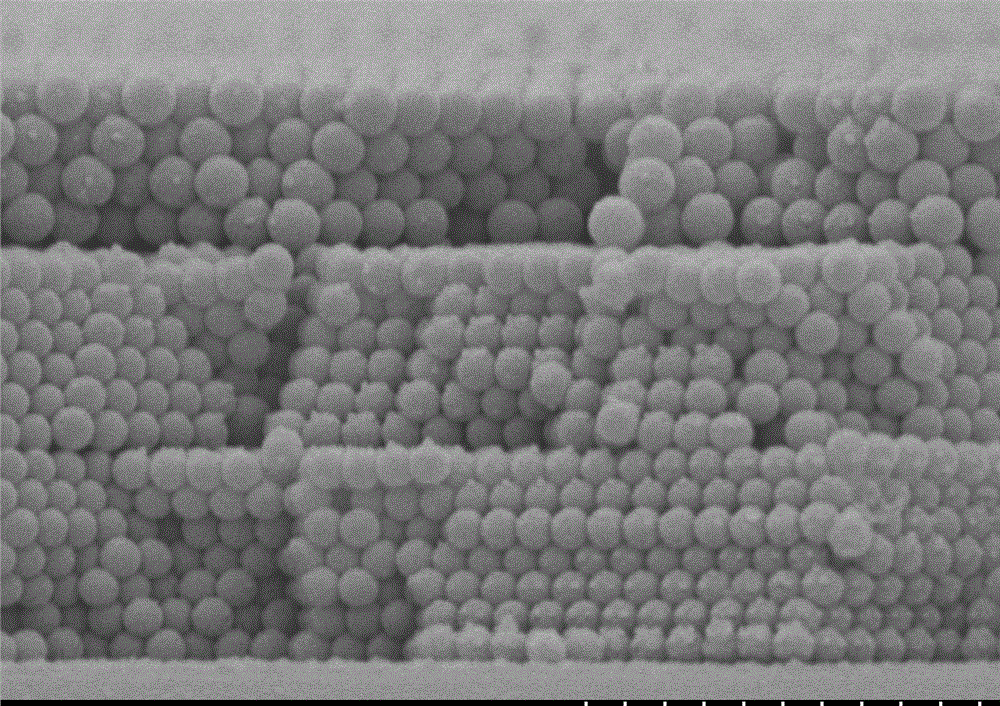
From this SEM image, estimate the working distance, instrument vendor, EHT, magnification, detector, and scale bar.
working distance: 12.3 mm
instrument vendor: Hitachi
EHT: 5 kV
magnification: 25 K X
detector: SE(M)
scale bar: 2000 nm